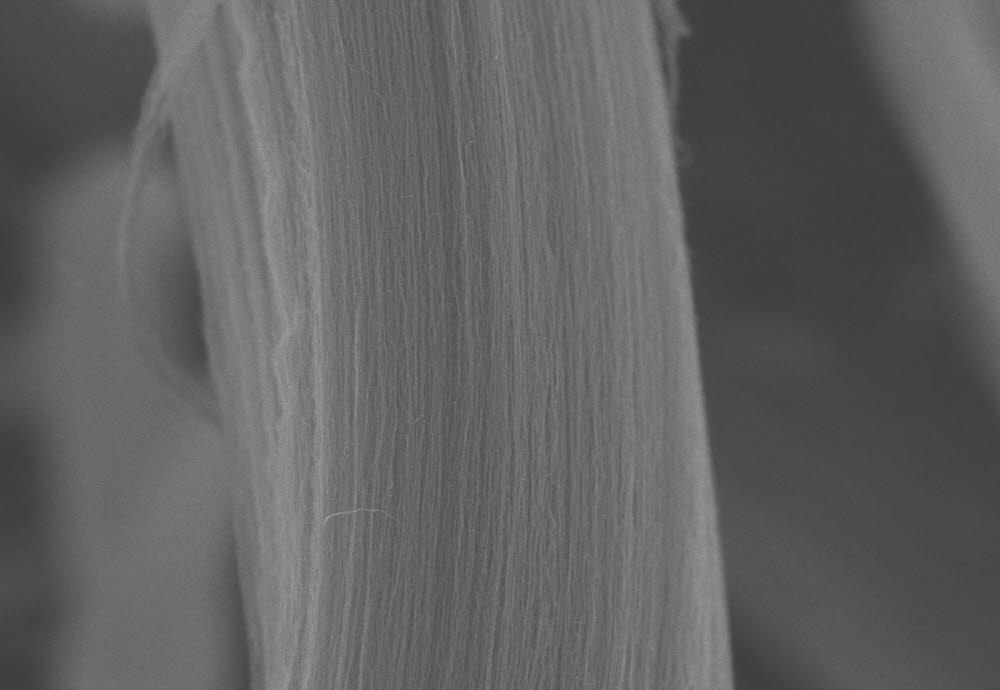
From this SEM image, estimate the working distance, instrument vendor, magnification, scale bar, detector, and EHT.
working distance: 7.8 mm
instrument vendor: Hitachi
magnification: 10 K X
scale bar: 5000 nm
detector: SE(U)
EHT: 5 kV